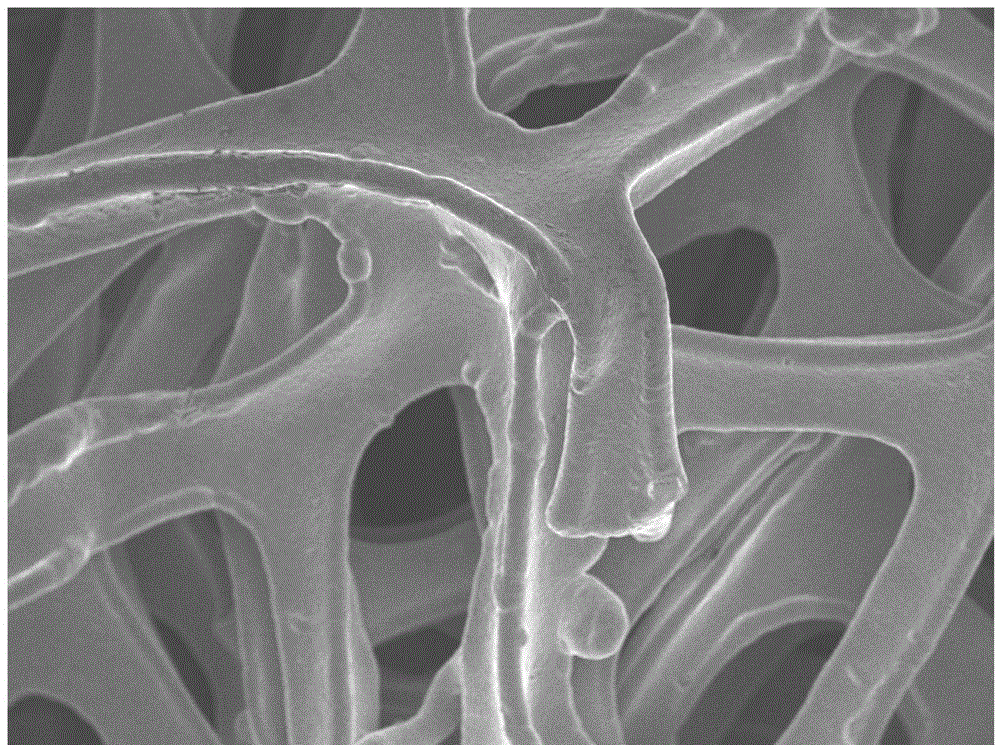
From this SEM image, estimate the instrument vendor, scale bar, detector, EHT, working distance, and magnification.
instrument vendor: JEOL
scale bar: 100000 nm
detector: SEI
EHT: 5 kV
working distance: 11.4 mm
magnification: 0.2 K X